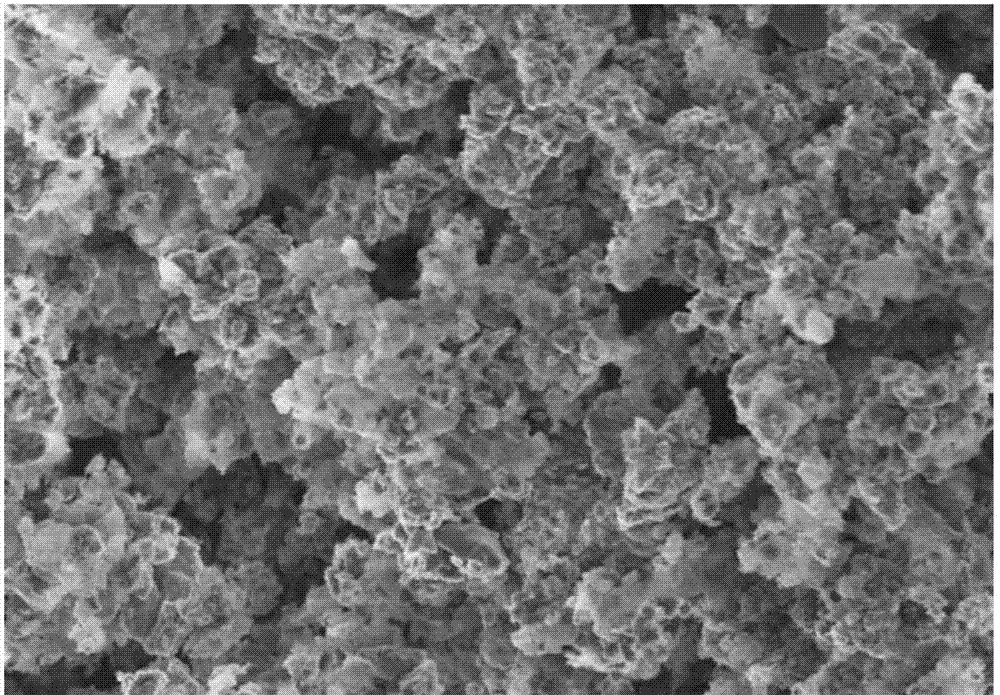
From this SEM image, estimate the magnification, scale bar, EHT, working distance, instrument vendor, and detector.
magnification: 10 K X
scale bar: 5000 nm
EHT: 2 kV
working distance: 4.8 mm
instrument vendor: Hitachi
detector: SE(M)